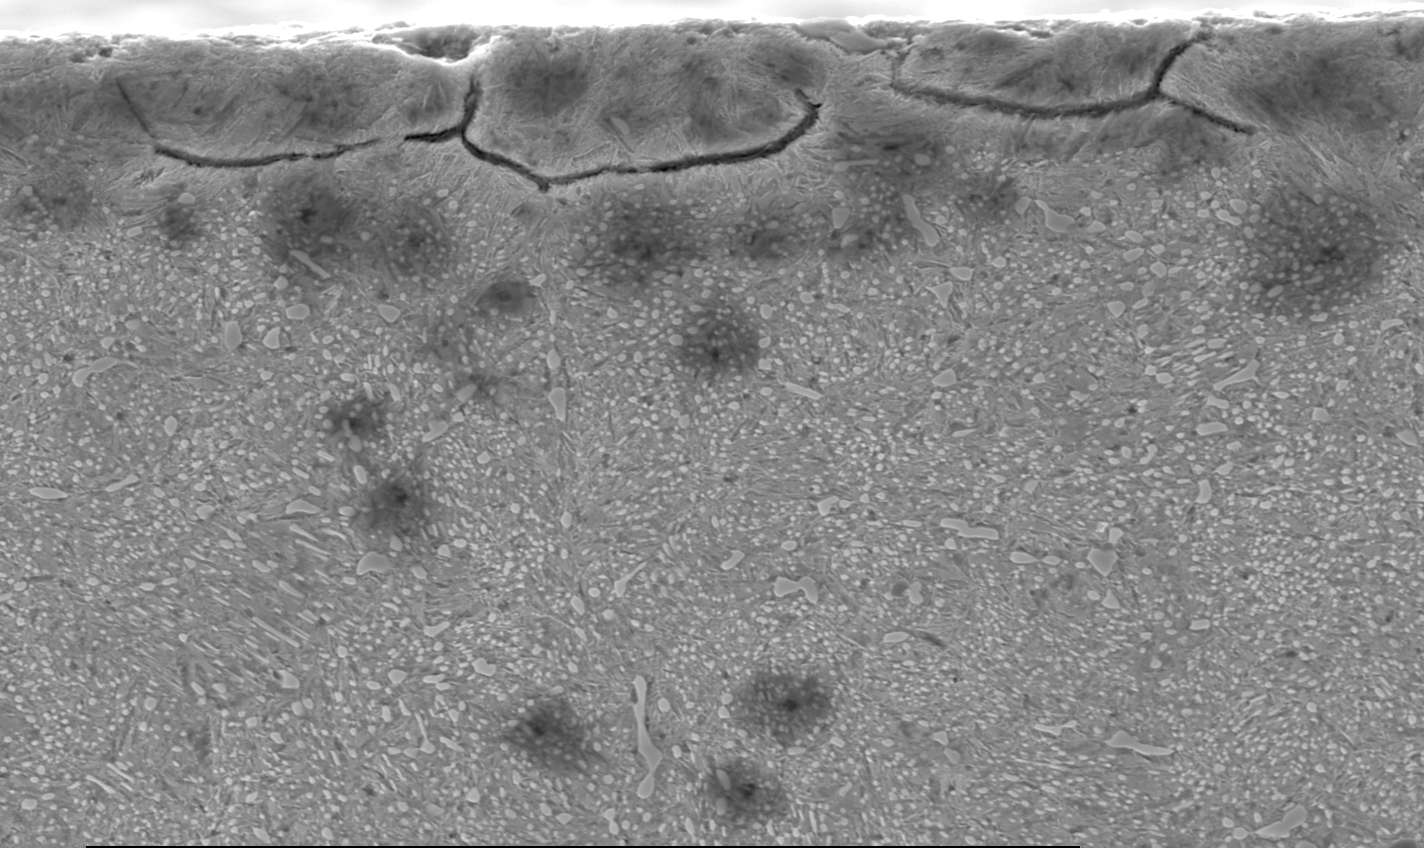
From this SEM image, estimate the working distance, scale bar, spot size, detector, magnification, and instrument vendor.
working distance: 10.6 mm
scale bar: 20000 nm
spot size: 5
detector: SE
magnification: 1.5 K X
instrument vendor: FEI